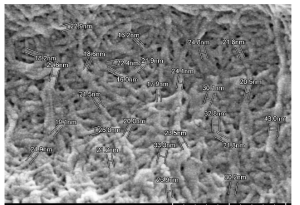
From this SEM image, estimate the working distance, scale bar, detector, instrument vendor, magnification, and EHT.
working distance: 6.1 mm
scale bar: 500 nm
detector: SE(U)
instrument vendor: Hitachi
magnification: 100 K X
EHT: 2 kV